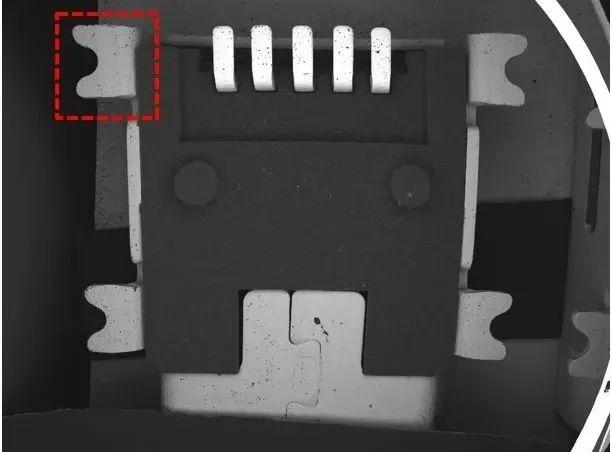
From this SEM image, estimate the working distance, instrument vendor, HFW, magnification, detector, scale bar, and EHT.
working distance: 15.22 mm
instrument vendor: Tescan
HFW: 13300 µm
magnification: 0.021 K X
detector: BSE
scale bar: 2000000 nm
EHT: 20 kV